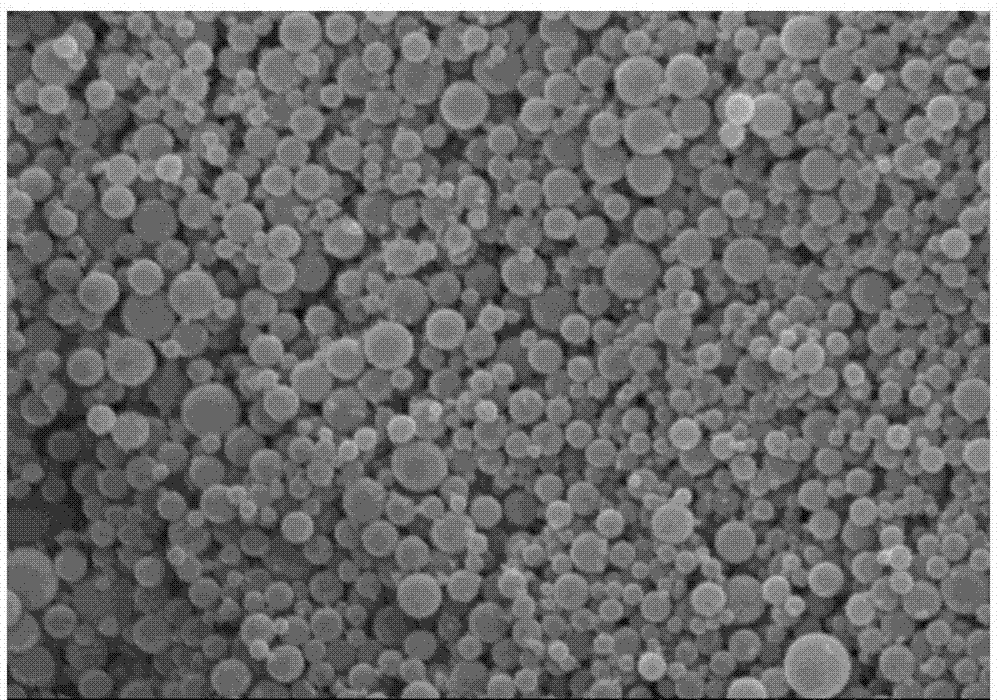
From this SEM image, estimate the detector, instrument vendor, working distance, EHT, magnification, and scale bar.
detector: SE(U)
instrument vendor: Hitachi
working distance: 6.2 mm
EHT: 15 kV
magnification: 5 K X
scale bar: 10000 nm